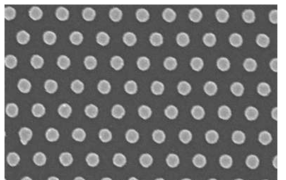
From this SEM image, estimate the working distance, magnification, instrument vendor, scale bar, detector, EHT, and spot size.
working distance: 4.7 mm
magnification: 100 K X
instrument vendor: FEI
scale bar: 500 nm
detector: TLD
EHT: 10 kV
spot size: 3